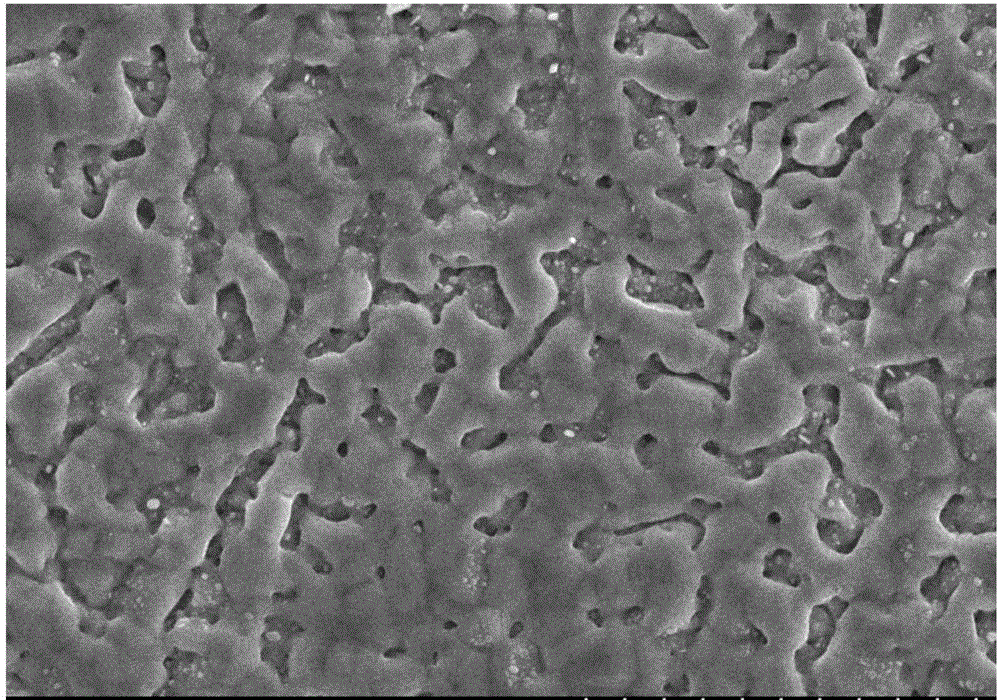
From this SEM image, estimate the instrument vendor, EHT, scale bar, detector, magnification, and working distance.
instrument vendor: Hitachi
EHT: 15 kV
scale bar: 10000 nm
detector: SE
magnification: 5 K X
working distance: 10.9 mm